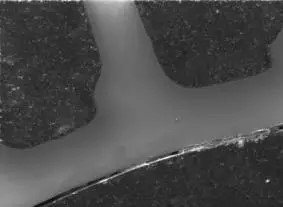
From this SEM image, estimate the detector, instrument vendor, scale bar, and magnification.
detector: SEI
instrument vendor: JEOL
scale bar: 1000000 nm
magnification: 0.0211 K X